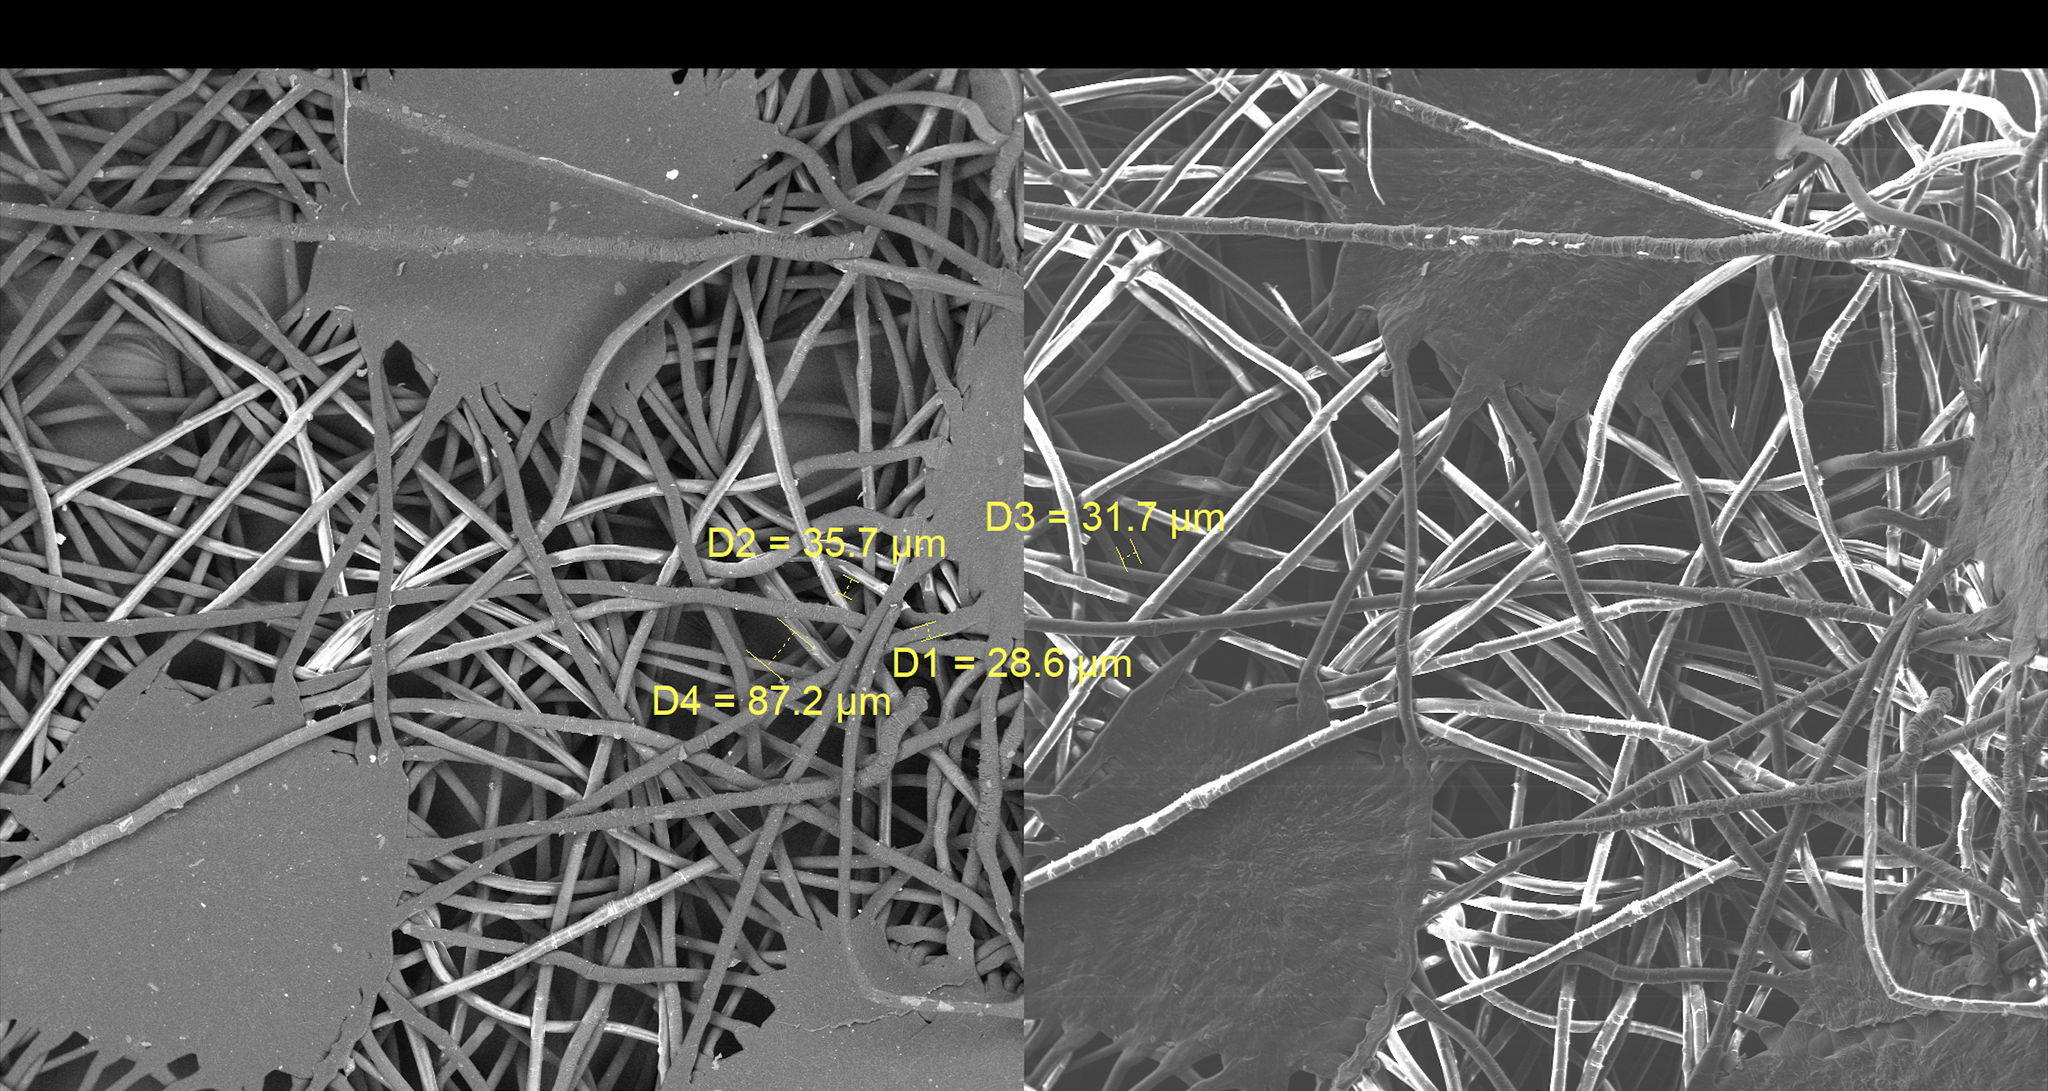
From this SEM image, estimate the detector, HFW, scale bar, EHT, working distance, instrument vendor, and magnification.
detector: LE-BSE, SE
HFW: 1990 µm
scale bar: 1000000 nm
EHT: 10 kV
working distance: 15 mm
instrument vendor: Tescan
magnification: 0.104 K X